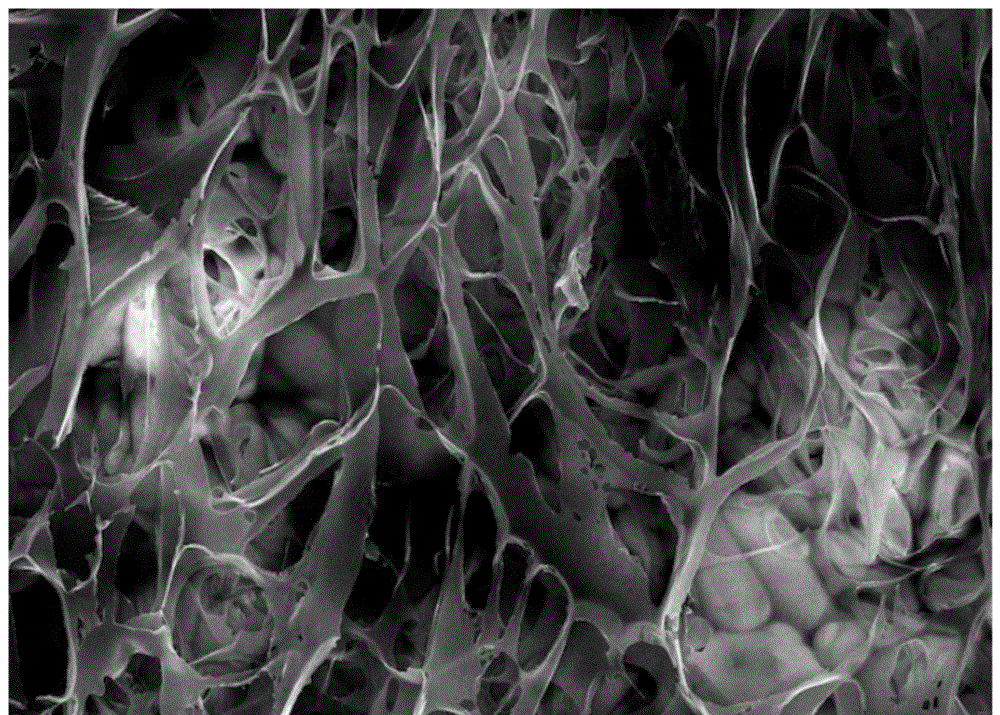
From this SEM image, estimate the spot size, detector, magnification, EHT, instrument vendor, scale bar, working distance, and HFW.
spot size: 6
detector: ETD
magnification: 0.65 K X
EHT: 30 kV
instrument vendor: Thermo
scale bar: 100000 nm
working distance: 10 mm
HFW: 638 µm